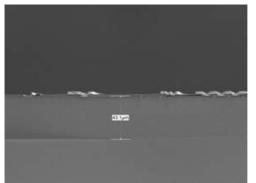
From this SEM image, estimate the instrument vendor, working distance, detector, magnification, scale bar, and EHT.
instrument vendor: JEOL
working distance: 10 mm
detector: LED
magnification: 0.5 K X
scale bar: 10000 nm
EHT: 5 kV